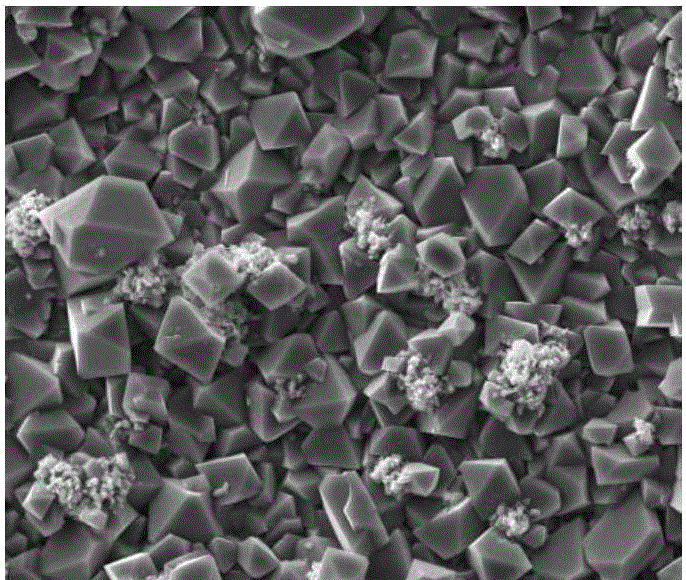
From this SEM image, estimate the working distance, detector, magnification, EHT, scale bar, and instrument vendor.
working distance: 5.2 mm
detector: ETD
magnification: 3 K X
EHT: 15 kV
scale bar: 30000 nm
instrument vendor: FEI